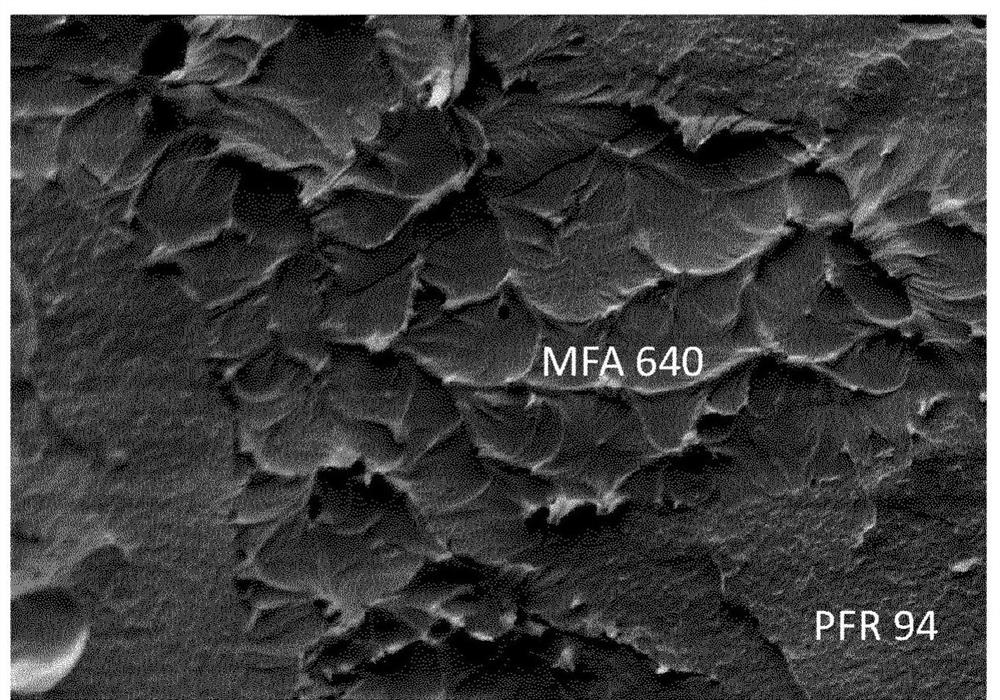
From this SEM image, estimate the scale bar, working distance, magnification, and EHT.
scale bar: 50000 nm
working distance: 14 mm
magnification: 0.5 K X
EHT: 15 kV